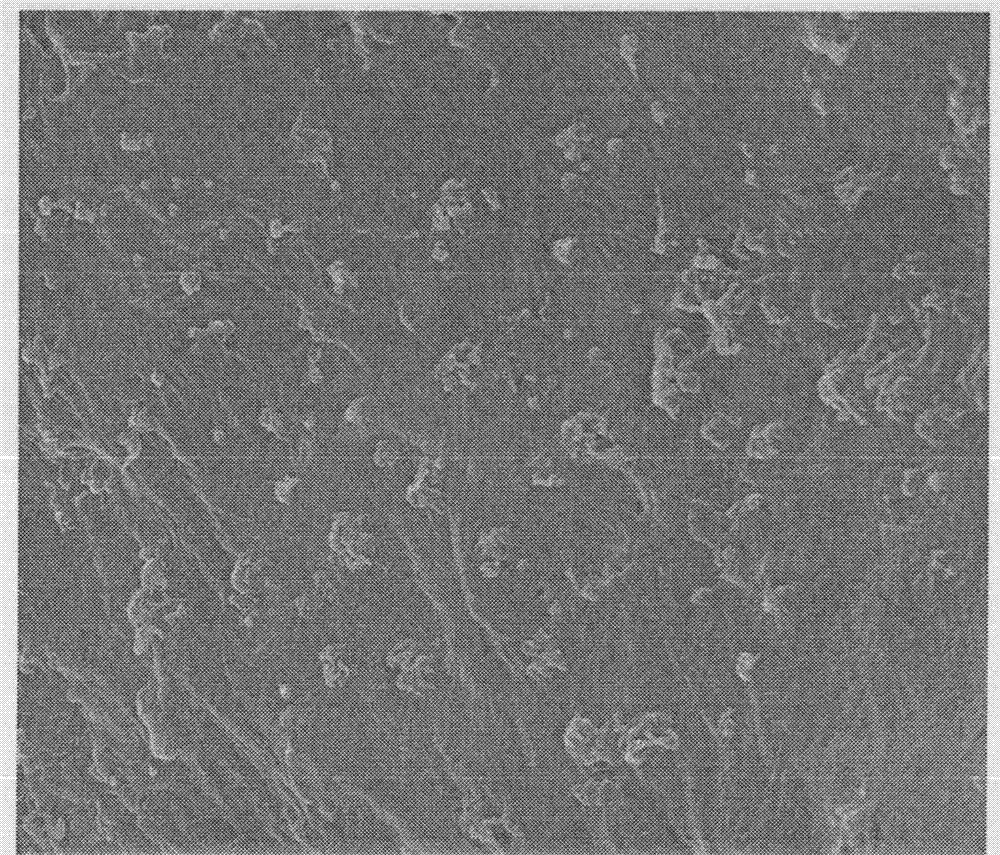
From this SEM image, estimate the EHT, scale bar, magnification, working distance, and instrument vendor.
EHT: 20 kV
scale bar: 3000 nm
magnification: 15 K X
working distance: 12.2 mm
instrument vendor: Hitachi